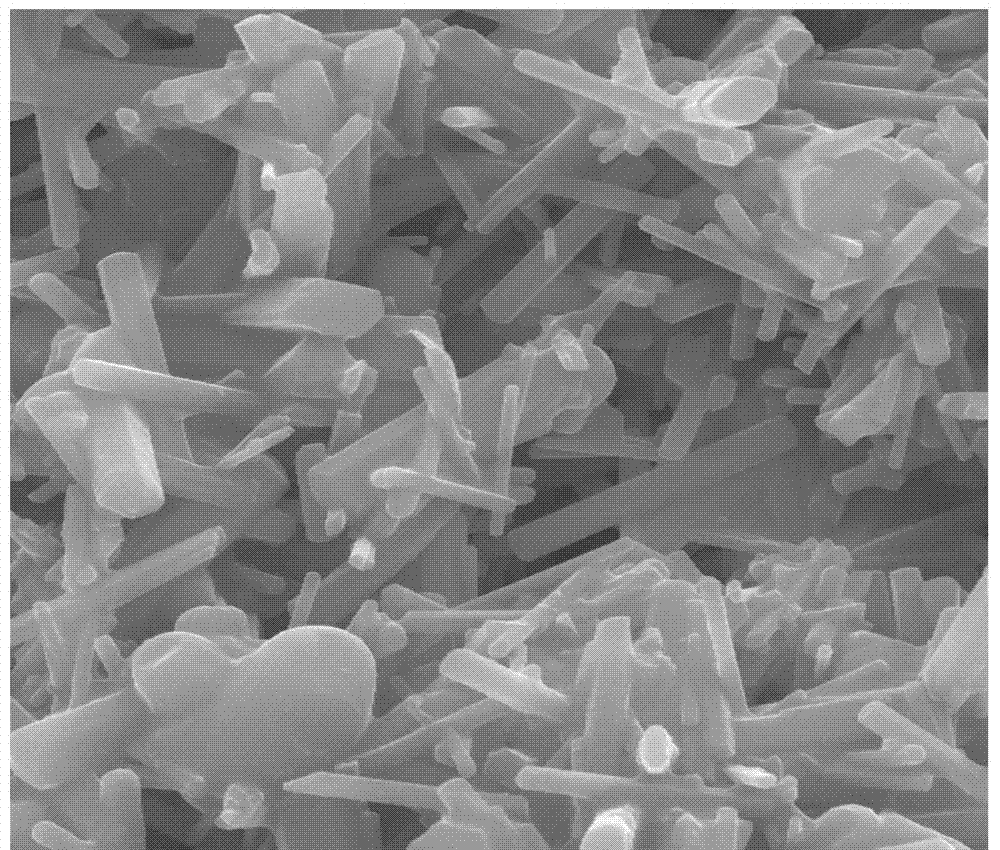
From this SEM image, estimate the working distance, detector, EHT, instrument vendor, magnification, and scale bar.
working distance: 10.6 mm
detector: ETD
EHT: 20 kV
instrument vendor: FEI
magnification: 20 K X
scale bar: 5000 nm